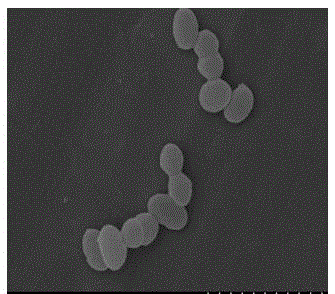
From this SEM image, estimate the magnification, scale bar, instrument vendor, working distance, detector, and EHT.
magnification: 15 K X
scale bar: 3000 nm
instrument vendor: Hitachi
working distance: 8.2 mm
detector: SE(M)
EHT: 15 kV